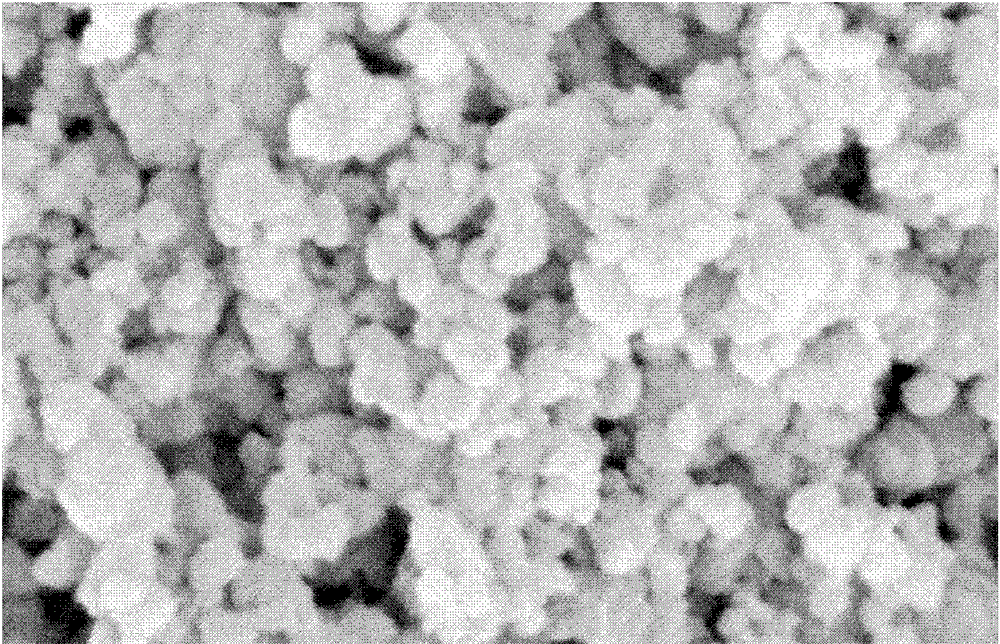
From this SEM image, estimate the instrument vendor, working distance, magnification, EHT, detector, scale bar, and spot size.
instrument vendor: FEI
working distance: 5.1 mm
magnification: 20 K X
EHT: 20 kV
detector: SE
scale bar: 1000 nm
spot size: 2.5